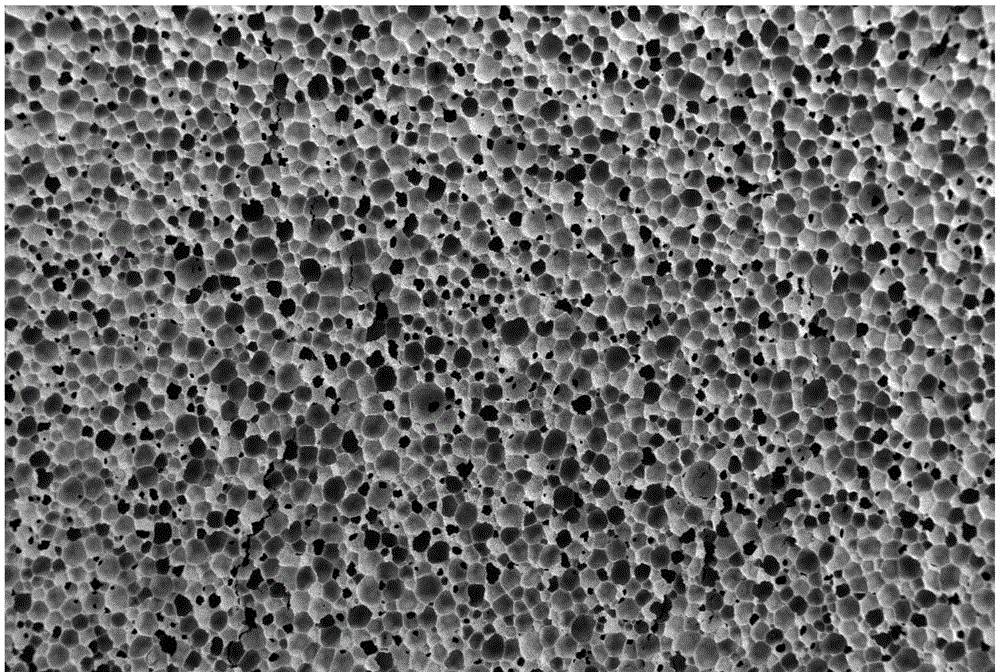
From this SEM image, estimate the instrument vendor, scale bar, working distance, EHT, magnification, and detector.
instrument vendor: Zeiss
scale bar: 100000 nm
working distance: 7.2 mm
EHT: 8 kV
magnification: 0.031 K X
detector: SE2